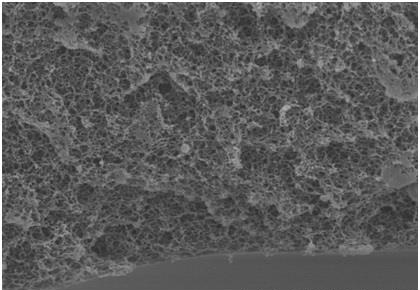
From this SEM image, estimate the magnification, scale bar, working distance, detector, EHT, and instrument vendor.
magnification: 5 K X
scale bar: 10000 nm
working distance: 9.4 mm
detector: SE(M)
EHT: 7 kV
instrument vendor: Hitachi